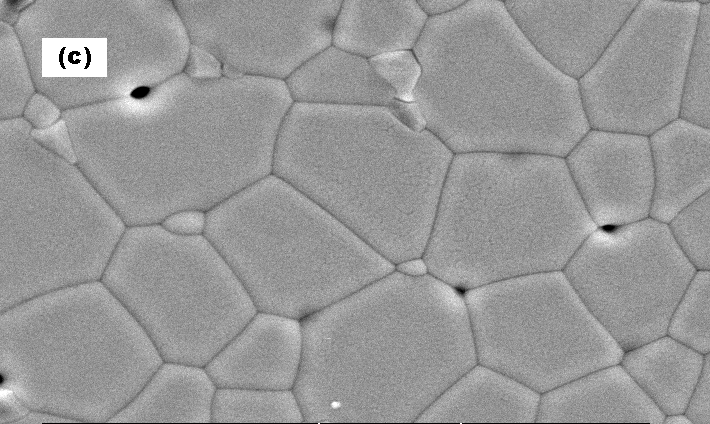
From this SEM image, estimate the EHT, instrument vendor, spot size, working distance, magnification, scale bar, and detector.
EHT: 20 kV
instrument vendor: FEI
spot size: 4.2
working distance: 7 mm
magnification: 5 K X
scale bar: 5000 nm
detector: SE